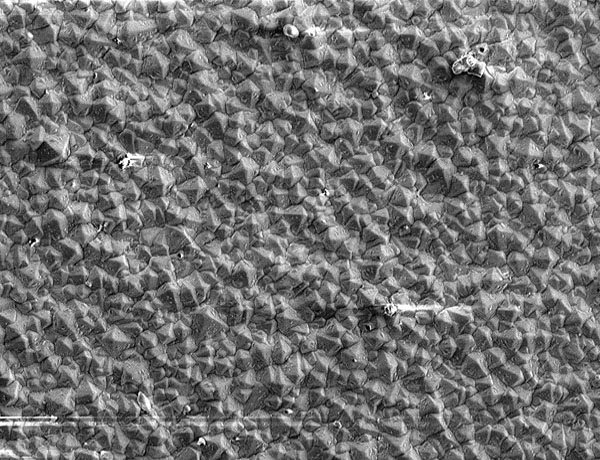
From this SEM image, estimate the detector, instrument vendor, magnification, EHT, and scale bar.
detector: SEI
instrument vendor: JEOL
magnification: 1 K X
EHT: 5 kV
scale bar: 10000 nm